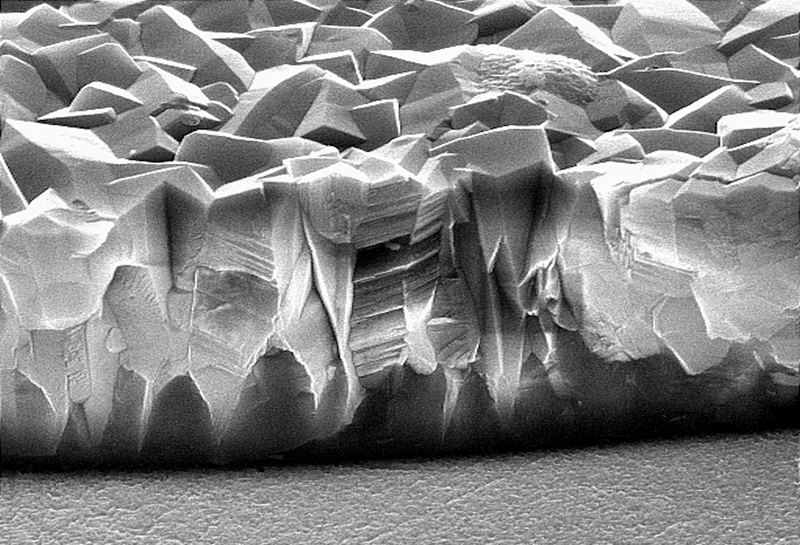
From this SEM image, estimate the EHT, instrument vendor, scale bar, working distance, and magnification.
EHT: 15 kV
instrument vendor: JEOL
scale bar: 2000 nm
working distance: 7 mm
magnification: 10 K X